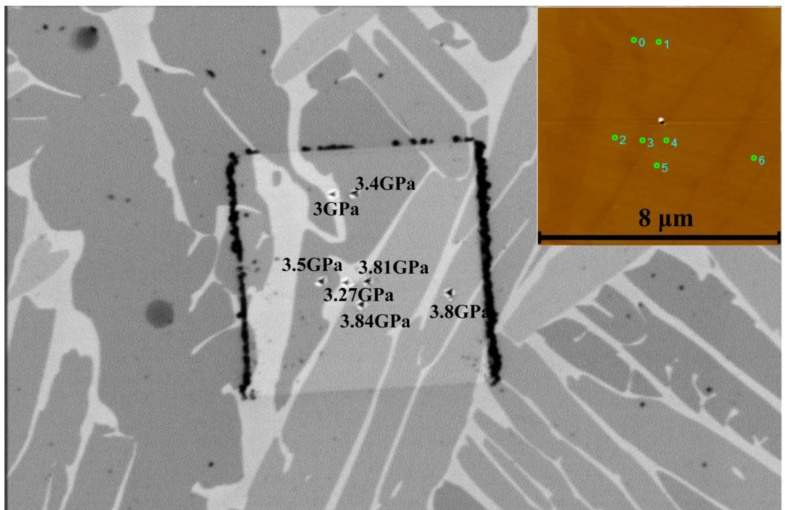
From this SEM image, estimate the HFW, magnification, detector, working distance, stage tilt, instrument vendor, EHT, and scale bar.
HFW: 25.4 µm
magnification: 5 K X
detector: CBS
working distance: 4 mm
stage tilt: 0°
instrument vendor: FEI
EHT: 5 kV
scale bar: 5000 nm